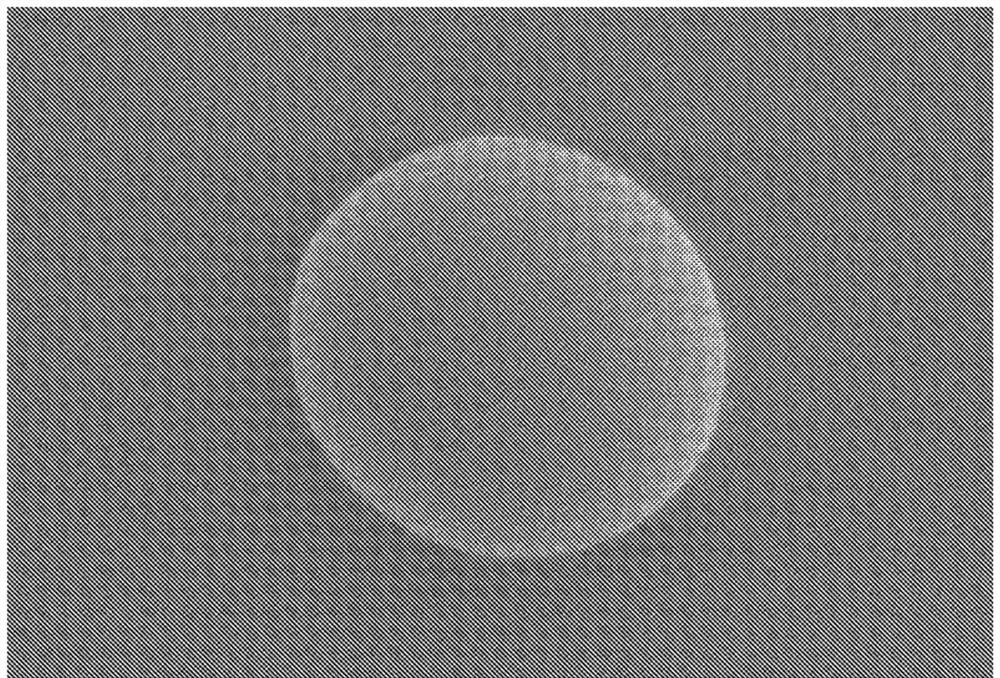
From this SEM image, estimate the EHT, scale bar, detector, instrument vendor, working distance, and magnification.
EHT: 1 kV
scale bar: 10000 nm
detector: SE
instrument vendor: Hitachi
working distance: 9.6 mm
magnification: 3 K X